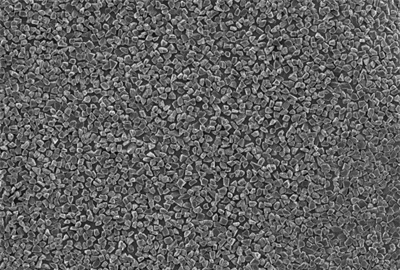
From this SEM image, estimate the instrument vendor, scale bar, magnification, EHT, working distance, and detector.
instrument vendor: JEOL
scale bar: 10000 nm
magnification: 0.5 K X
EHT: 10 kV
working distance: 10.1 mm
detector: SEI